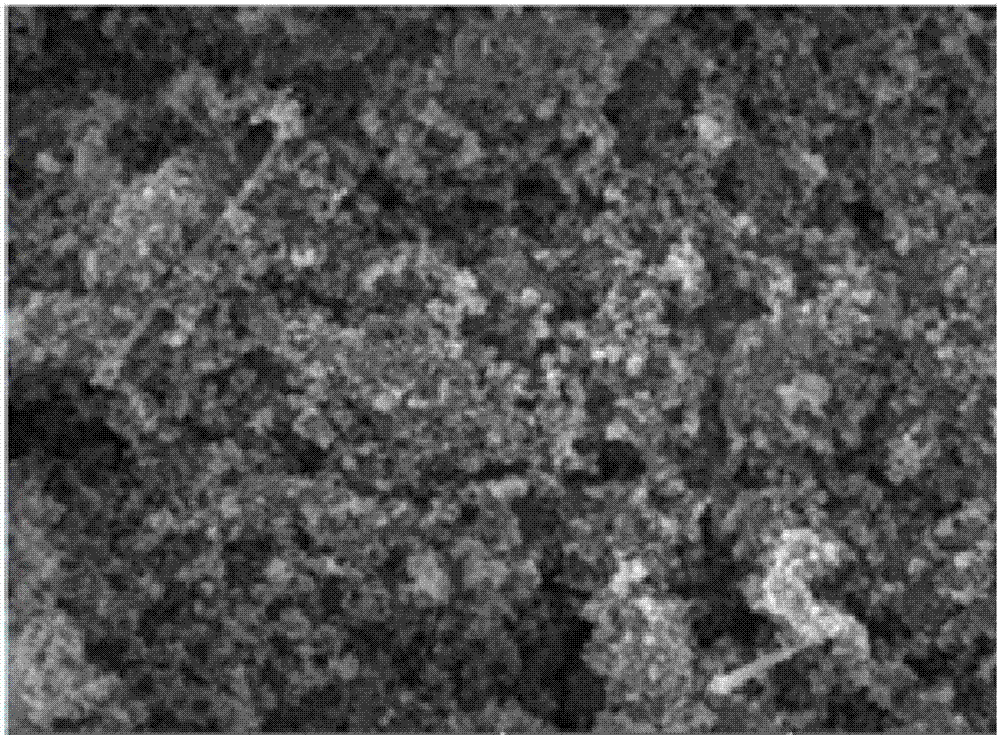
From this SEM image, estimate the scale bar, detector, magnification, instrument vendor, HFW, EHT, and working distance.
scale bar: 5000 nm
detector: SE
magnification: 12.1 K X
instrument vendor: Tescan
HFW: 17.2 µm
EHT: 30 kV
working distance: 9.89 mm